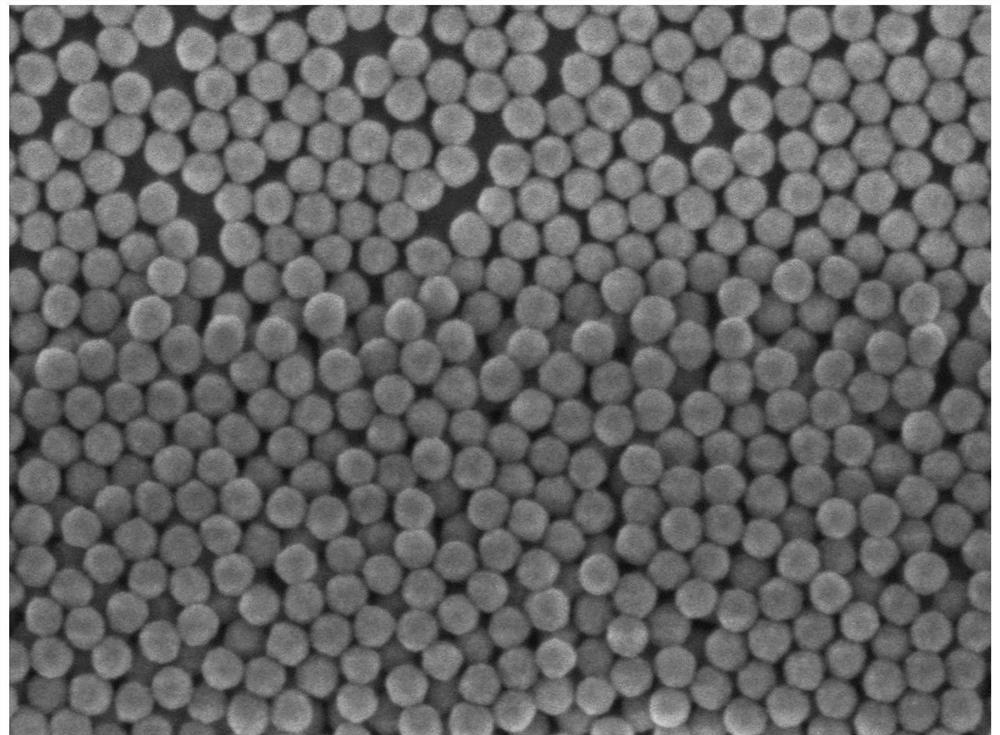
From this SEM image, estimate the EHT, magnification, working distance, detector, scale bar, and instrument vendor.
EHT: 1 kV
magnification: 100 K X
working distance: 3 mm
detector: UED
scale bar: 100 nm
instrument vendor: JEOL